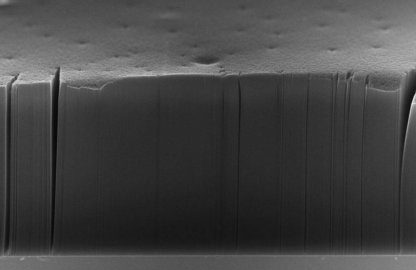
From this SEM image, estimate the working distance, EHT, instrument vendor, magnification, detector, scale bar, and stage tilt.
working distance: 4 mm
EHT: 15 kV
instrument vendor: Zeiss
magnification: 0.5 K X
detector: InLens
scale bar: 100000 nm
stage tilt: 70°